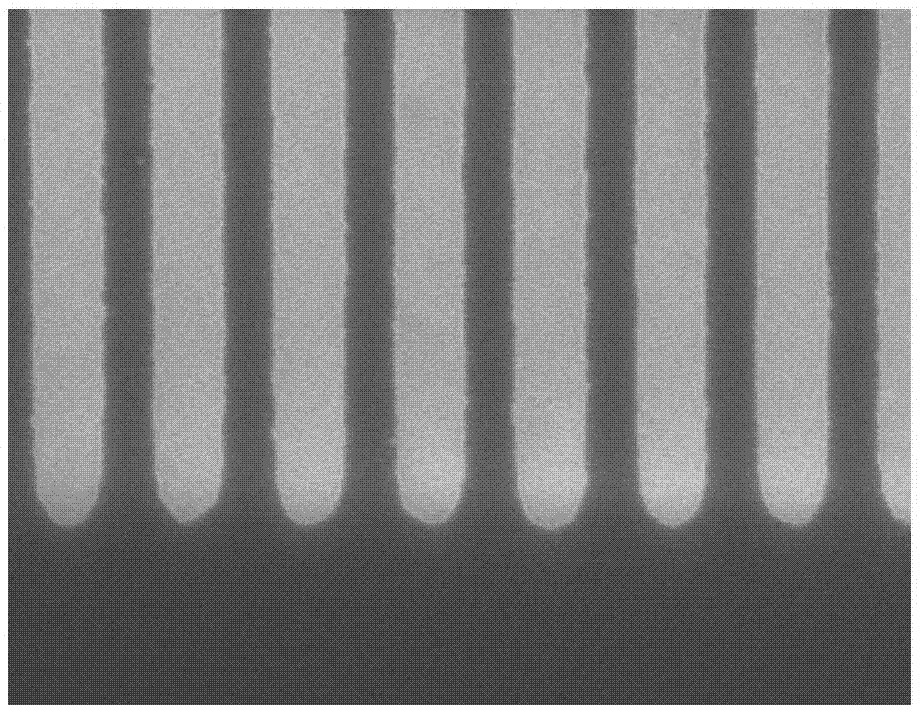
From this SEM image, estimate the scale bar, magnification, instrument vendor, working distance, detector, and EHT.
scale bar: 1000 nm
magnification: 50 K X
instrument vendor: Hitachi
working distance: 12.8 mm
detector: SE(U)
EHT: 15 kV